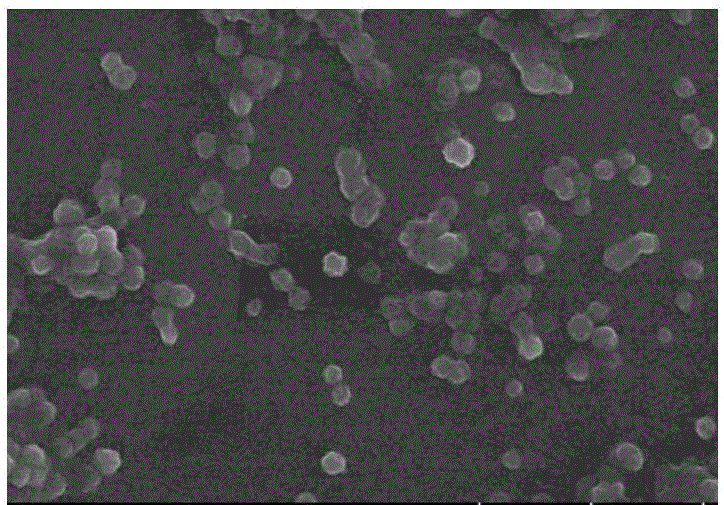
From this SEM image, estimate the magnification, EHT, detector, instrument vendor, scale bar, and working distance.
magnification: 20 K X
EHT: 5 kV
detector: SE(UL)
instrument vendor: Hitachi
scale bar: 2000 nm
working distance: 10 mm